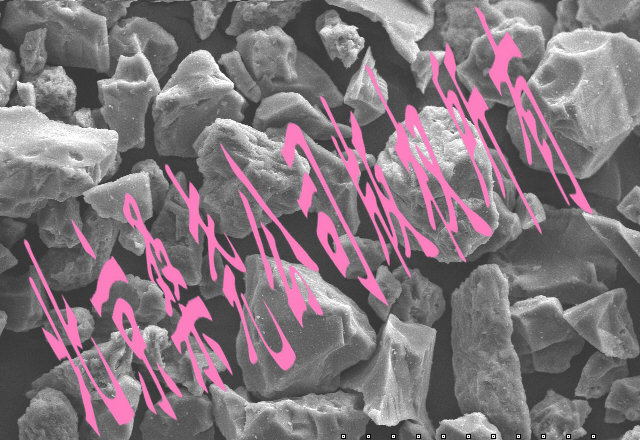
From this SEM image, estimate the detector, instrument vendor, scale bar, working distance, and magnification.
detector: SE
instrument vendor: Hitachi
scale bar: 100000 nm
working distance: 8.1 mm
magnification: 0.5 K X